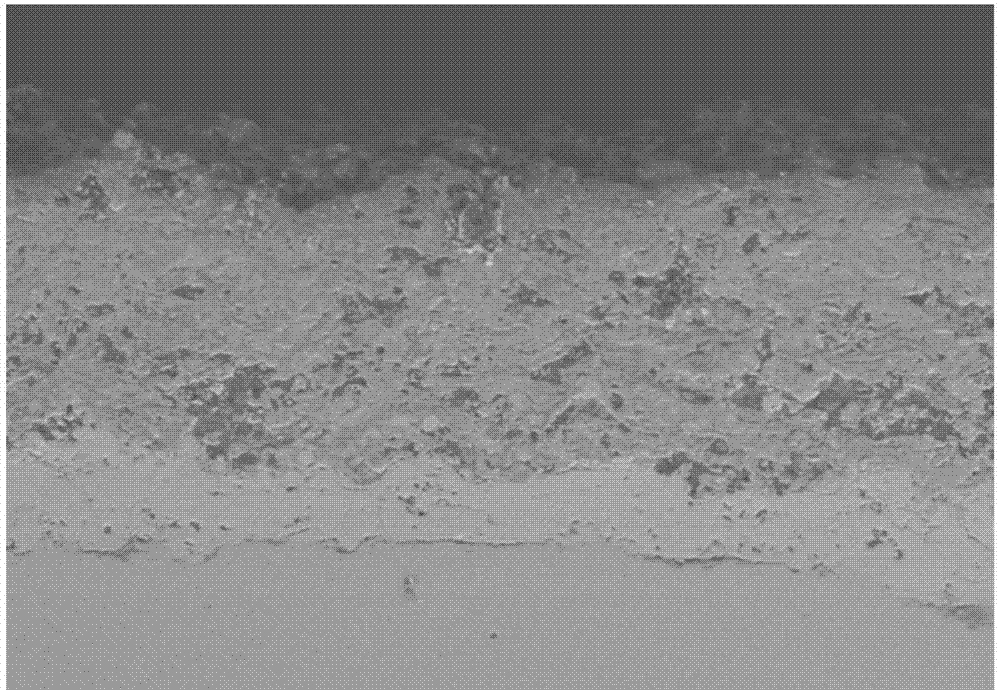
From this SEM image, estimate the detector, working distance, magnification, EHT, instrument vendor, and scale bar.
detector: SE(M)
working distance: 15 mm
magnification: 0.2 K X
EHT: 15 kV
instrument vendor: Hitachi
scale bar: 200000 nm